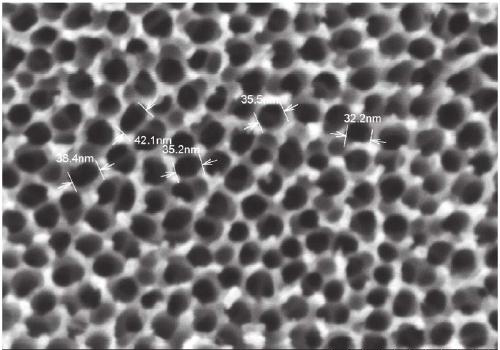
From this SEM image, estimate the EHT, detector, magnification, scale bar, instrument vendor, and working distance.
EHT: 5 kV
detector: SE(M)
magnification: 200 K X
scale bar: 200 nm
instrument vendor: Hitachi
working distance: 9.2 mm